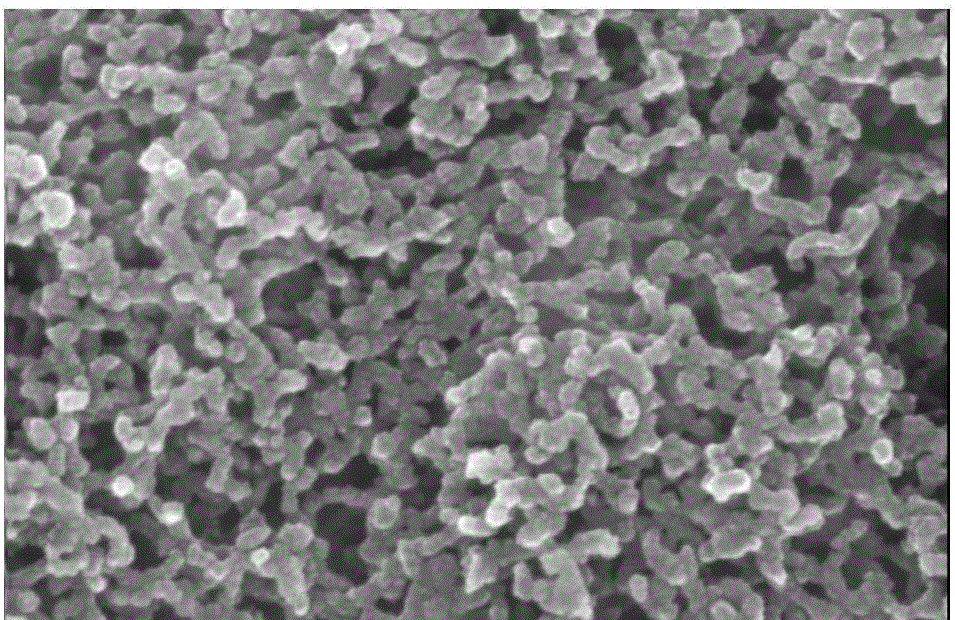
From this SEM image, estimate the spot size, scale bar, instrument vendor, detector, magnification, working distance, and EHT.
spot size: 4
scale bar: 200 nm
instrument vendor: FEI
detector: TLD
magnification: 200 K X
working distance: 6.7 mm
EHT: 20 kV